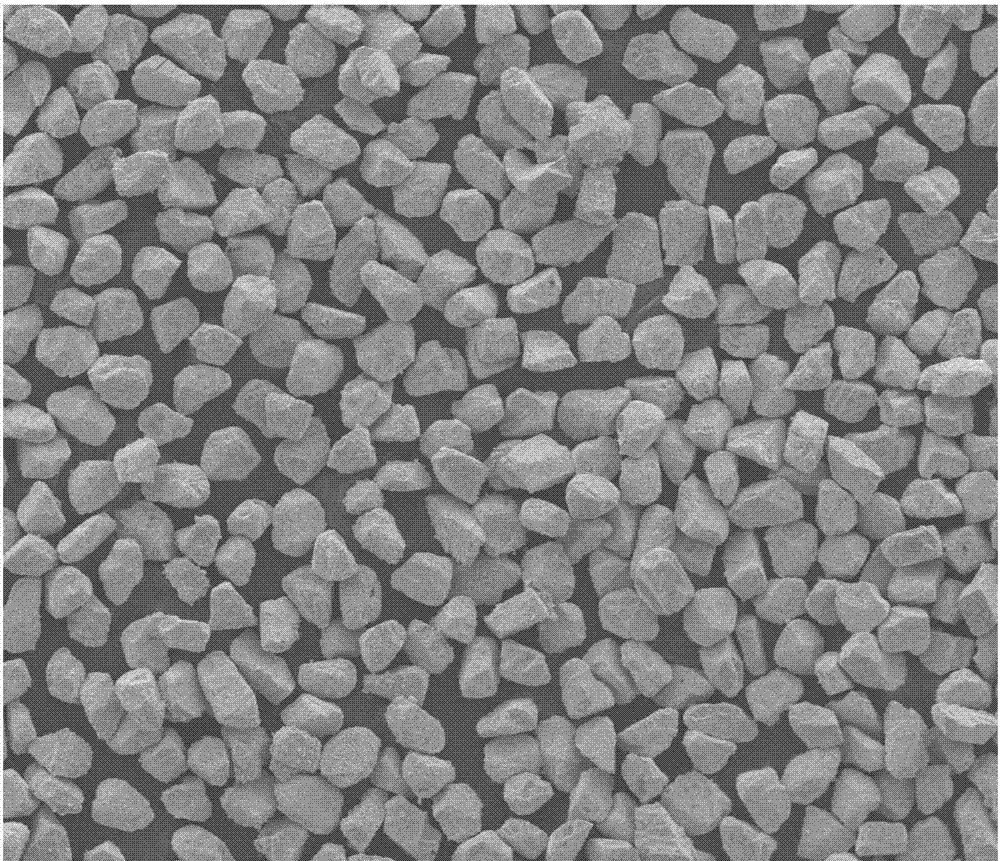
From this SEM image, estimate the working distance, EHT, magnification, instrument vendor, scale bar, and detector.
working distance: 10 mm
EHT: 20 kV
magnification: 0.25 K X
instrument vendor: FEI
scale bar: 400000 nm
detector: ETD